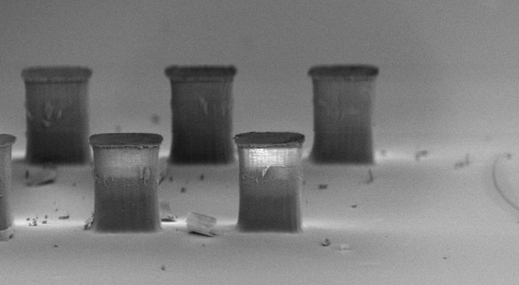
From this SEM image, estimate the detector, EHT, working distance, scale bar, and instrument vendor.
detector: InLens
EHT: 5 kV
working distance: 5 mm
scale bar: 20000 nm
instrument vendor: Zeiss